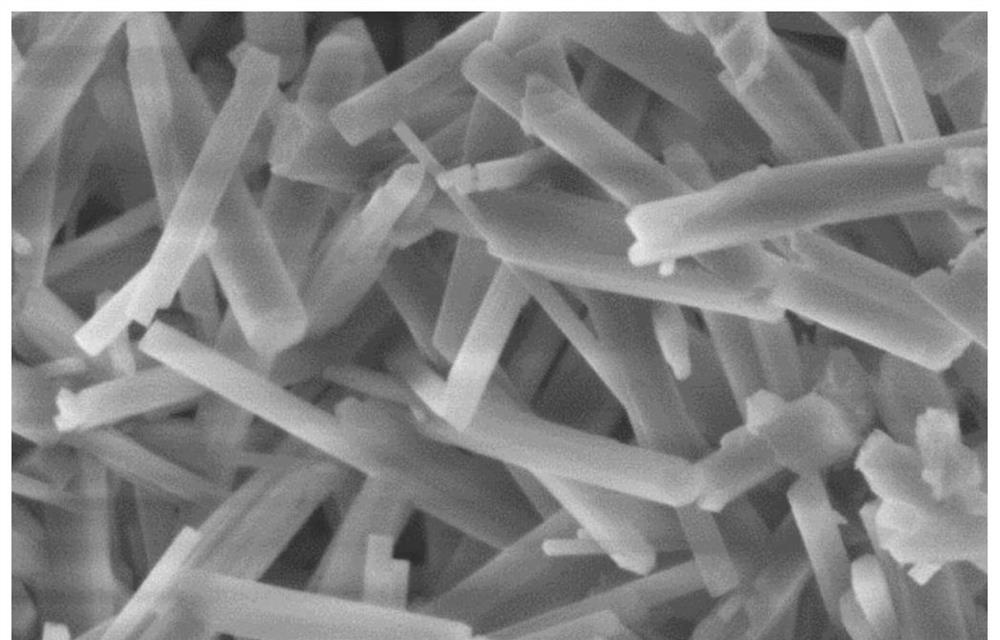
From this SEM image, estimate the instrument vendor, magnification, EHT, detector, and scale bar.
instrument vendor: Hitachi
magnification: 40 K X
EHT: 3 kV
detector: SE(U)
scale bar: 1000 nm